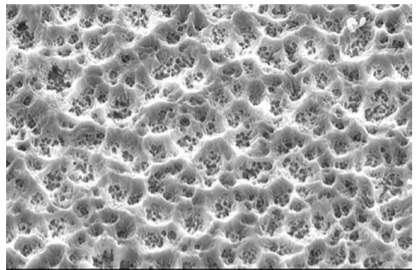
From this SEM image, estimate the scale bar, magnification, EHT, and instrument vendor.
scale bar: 10000 nm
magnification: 1 K X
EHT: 20 kV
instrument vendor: JEOL